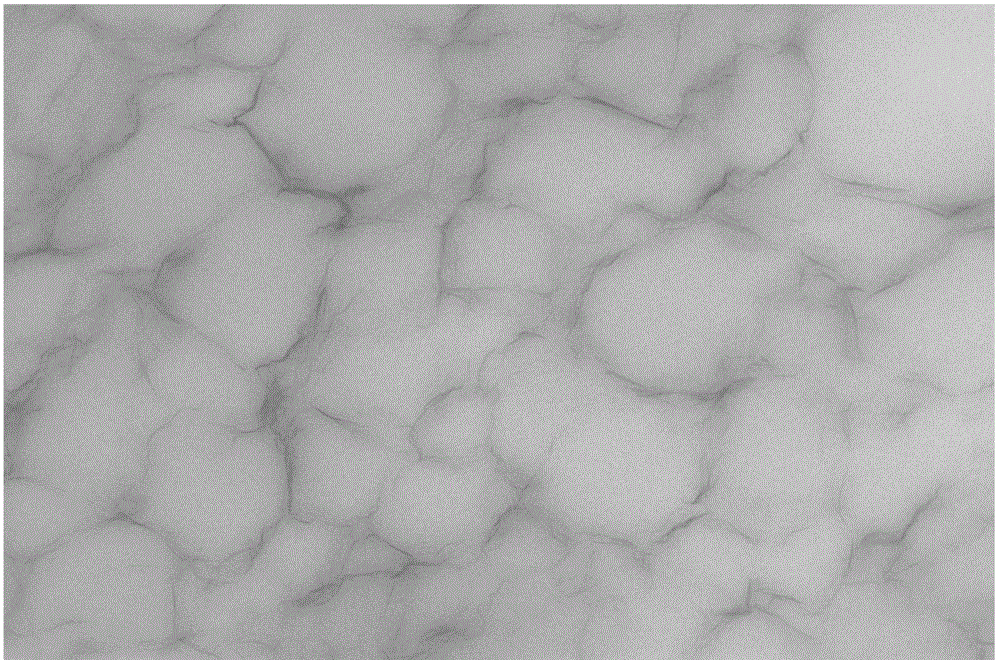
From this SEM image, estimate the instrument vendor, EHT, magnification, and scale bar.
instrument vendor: FEI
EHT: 200 kV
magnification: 9.9 K X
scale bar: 1000 nm